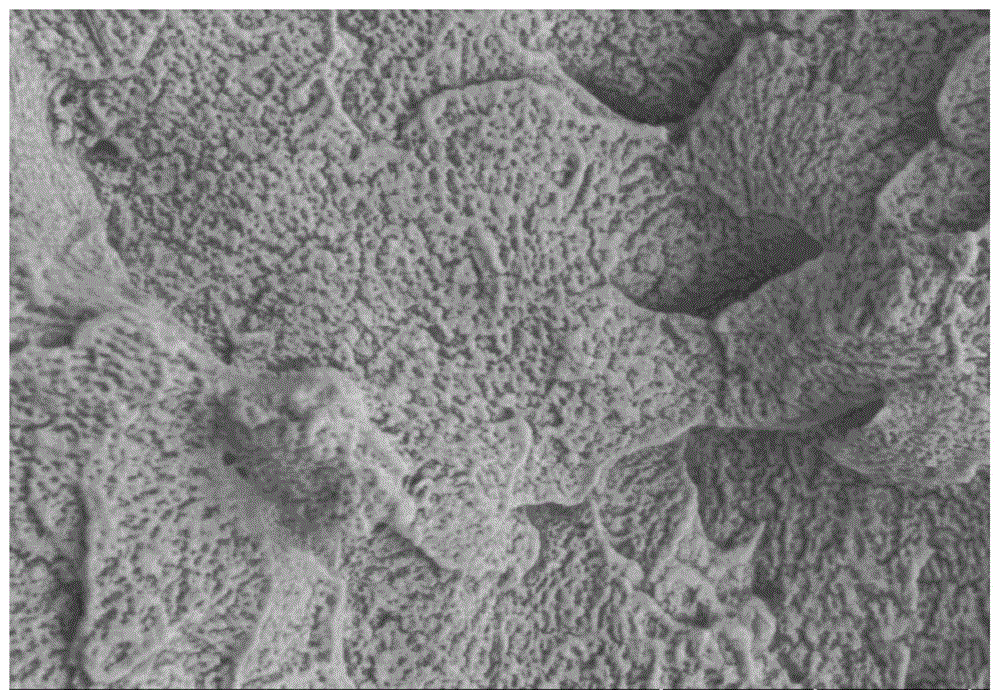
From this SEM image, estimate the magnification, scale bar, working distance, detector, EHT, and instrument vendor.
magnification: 20 K X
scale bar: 2000 nm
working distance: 11.4 mm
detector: SE(L)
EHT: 2 kV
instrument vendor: Hitachi